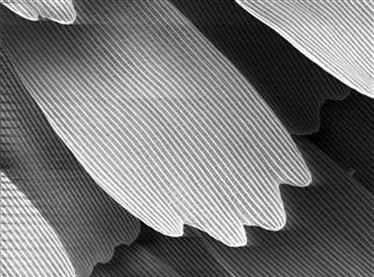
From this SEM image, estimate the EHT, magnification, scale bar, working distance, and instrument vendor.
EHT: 5 kV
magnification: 1 K X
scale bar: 20000 nm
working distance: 19 mm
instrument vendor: JEOL